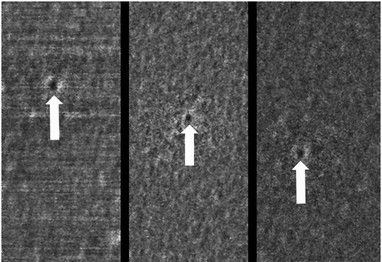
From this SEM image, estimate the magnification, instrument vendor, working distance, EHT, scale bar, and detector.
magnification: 100 K X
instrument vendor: Hitachi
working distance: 1.7 mm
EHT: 0.5 kV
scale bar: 500 nm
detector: SE+BSE(U)